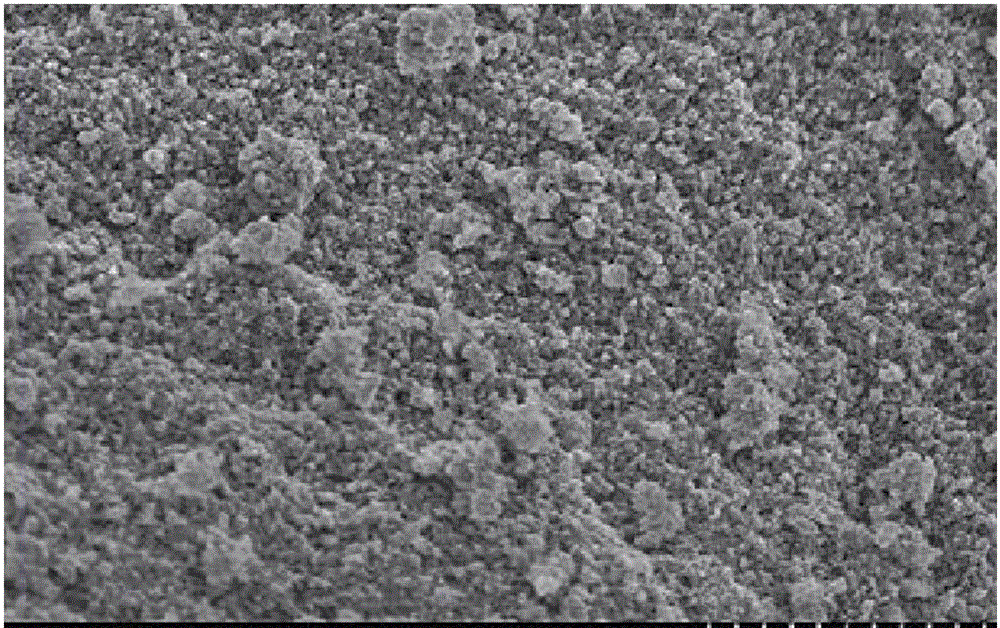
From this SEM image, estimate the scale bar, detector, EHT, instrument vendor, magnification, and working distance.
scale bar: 1000 nm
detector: SE(M)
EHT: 2 kV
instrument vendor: Hitachi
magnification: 35 K X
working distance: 5.7 mm